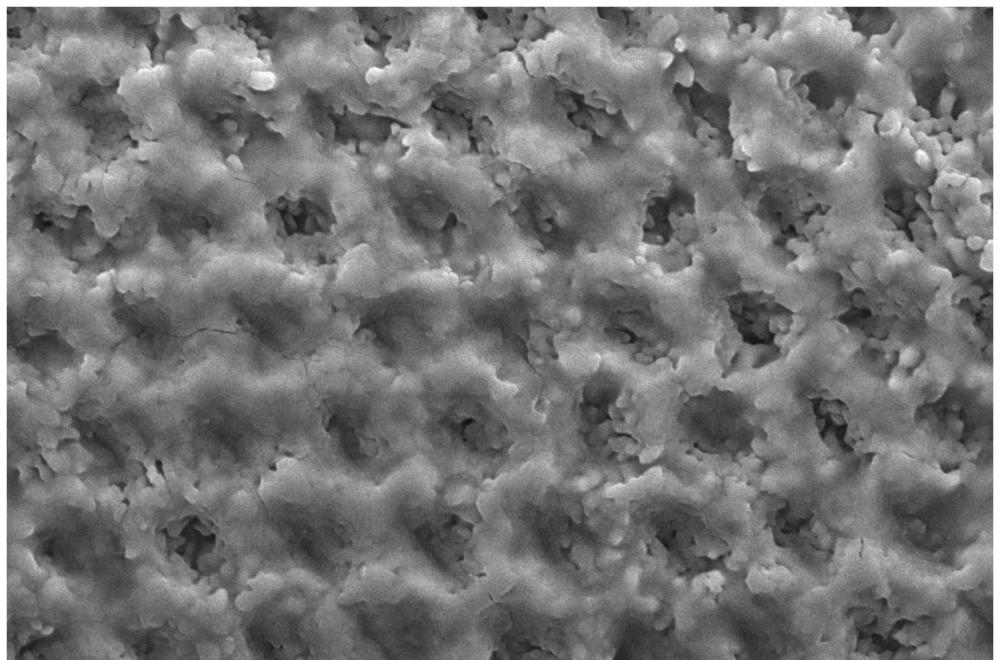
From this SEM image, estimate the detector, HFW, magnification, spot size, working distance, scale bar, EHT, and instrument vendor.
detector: ETD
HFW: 51.8 µm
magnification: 8 K X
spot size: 3.5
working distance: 10.3 mm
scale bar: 10000 nm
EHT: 15 kV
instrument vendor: FEI